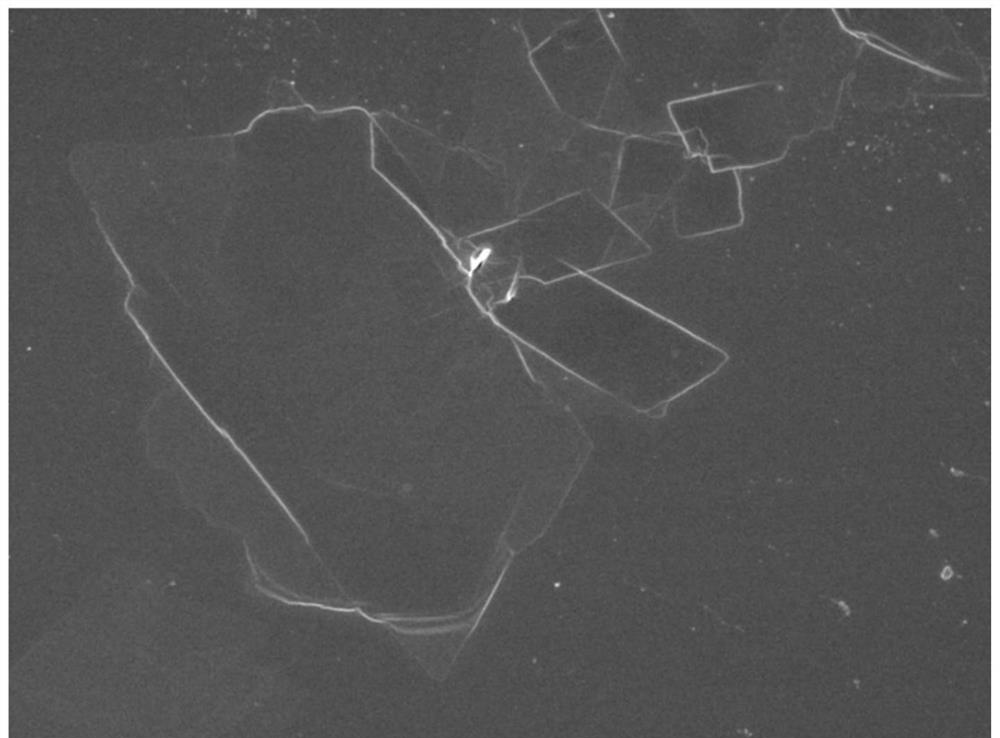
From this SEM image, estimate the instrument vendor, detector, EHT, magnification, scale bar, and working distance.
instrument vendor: JEOL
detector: SEI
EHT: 5 kV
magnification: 2.7 K X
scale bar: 10000 nm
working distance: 8.4 mm